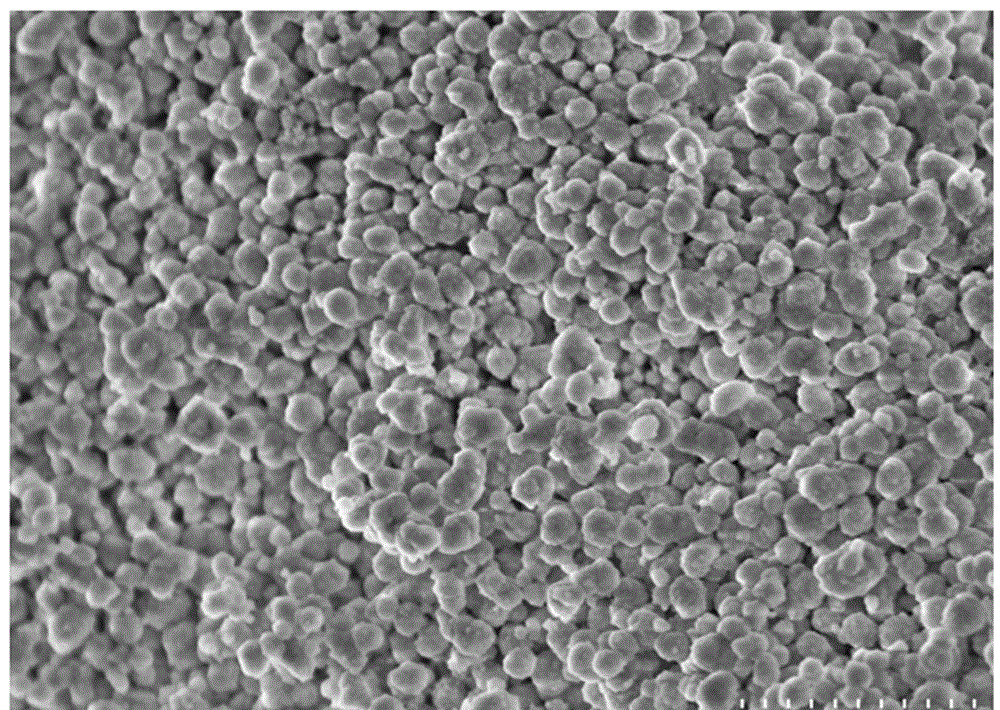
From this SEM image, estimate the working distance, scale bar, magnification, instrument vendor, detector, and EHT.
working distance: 3.6 mm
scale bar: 1000 nm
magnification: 30 K X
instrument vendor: Hitachi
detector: SE(M)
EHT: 3 kV